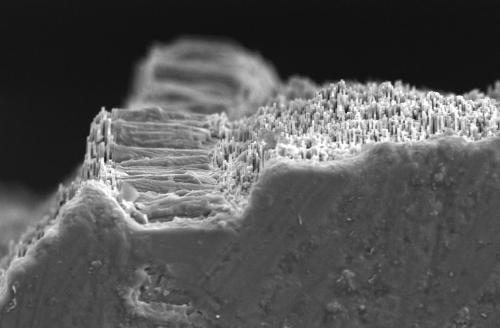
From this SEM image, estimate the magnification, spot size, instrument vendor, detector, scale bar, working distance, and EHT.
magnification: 1.75 K X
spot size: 3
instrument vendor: FEI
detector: ETD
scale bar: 50000 nm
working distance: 11.7 mm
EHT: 15 kV